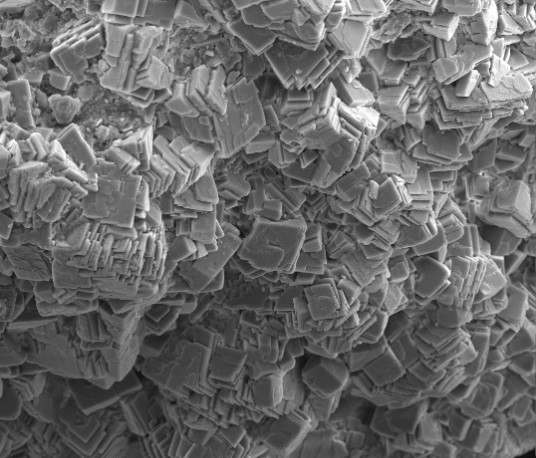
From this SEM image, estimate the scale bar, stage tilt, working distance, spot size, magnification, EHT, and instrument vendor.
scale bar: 100000 nm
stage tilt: -0.7°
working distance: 8 mm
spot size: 3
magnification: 0.928 K X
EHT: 20 kV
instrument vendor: FEI